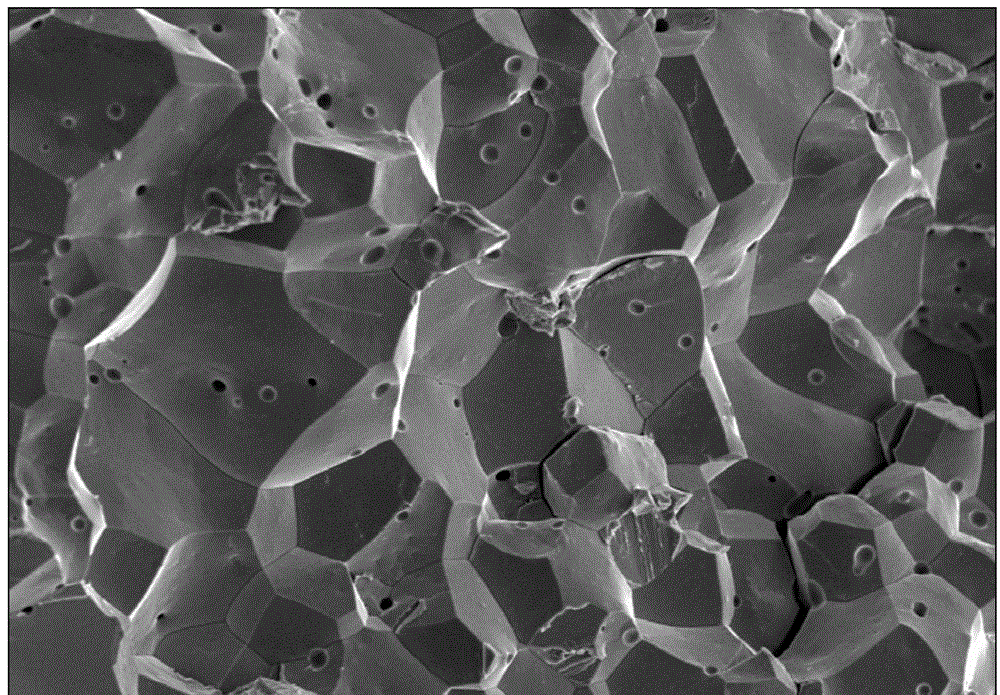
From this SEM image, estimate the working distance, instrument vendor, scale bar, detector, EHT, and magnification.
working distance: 13.3 mm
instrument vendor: Hitachi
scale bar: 100000 nm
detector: SE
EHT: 20 kV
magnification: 0.5 K X